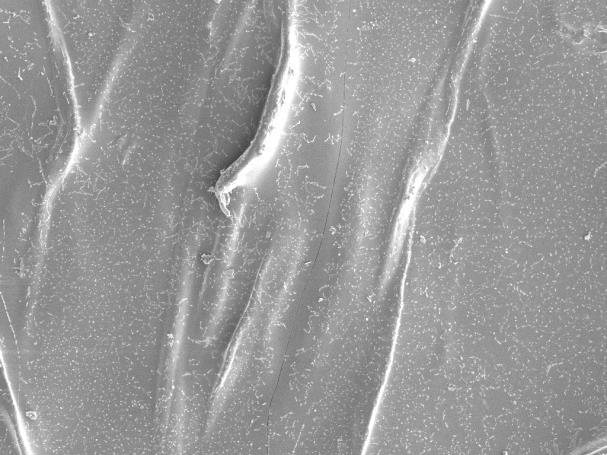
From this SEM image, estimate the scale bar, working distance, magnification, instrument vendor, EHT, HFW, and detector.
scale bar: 100000 nm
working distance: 9.92 mm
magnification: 0.6 K X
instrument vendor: Tescan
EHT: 5 kV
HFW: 461 µm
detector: SE